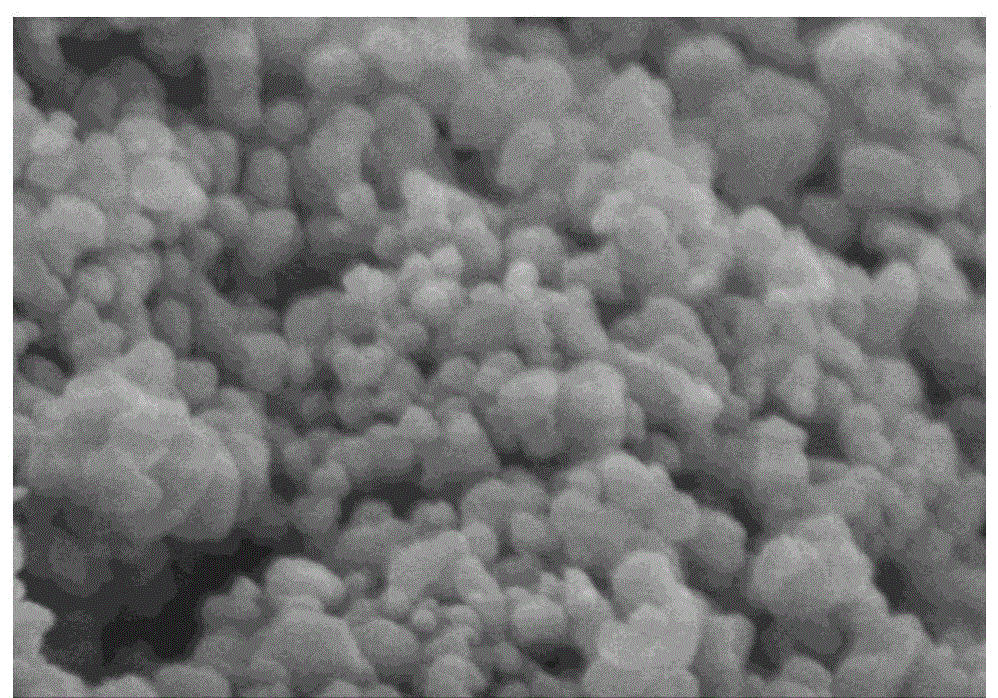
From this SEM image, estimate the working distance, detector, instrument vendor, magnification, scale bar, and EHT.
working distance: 10.3 mm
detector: SE(M)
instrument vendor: Hitachi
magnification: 60 K X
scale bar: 500 nm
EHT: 3 kV